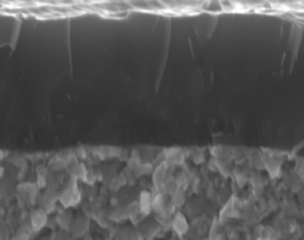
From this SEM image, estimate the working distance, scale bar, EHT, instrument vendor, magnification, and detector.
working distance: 6.1 mm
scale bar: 2000 nm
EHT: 15 kV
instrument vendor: Hitachi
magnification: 20 K X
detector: SE